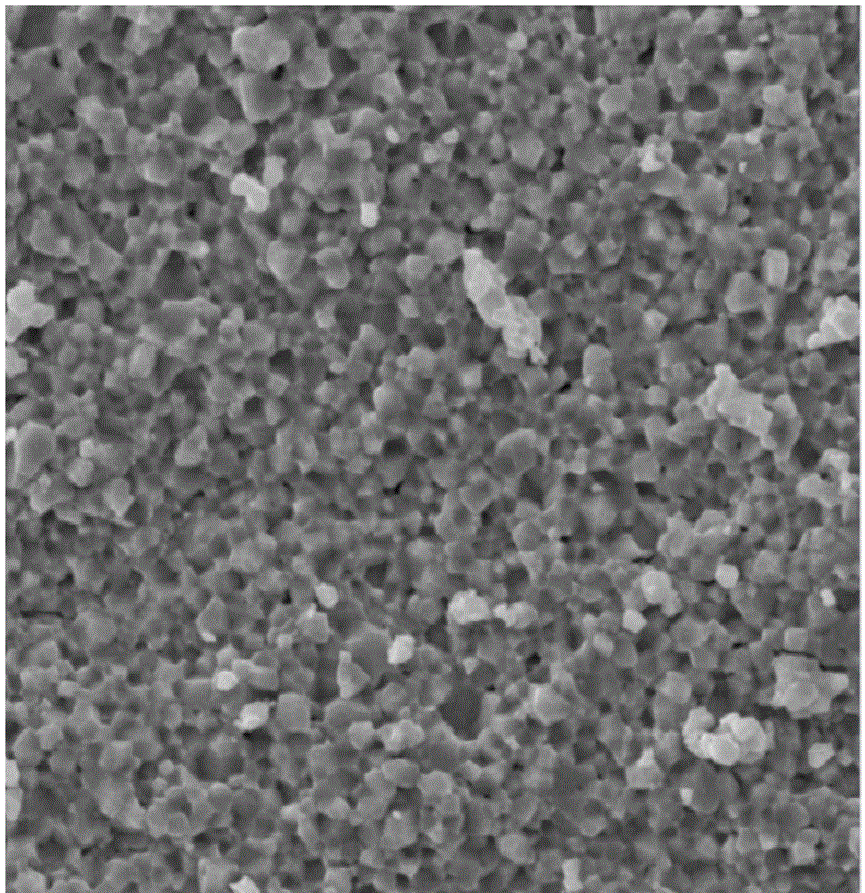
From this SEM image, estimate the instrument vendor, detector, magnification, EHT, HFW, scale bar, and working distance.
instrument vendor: Tescan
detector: SE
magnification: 5 K X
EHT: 20 kV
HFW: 44.9 µm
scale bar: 10000 nm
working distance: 14.98 mm